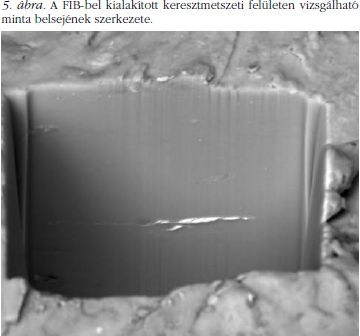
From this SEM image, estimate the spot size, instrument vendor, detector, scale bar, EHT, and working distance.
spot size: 7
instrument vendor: FEI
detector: BSED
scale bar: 5000 nm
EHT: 10 kV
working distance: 10 mm